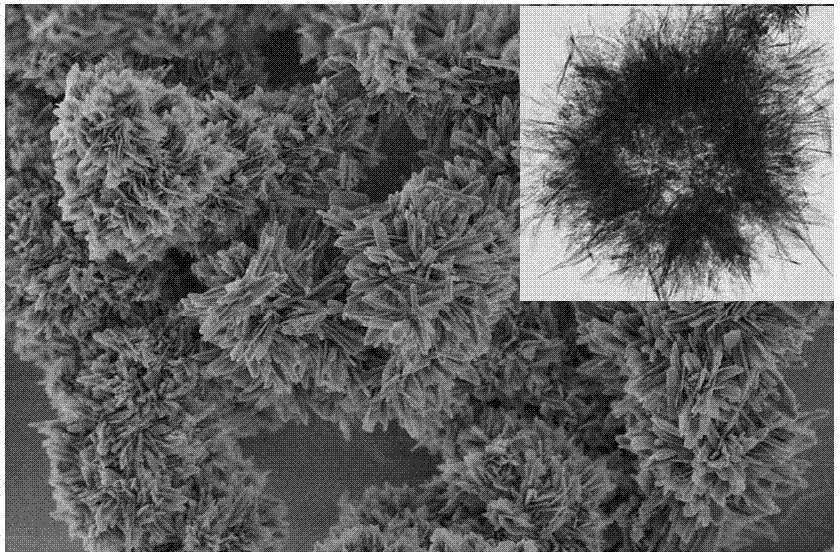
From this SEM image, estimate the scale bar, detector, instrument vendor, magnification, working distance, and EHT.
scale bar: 1000 nm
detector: TLD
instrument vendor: FEI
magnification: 100 K X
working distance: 2.9 mm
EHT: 1 kV